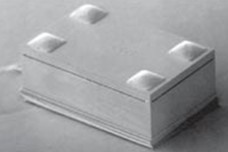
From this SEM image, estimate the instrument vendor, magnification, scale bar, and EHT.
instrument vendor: JEOL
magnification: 0.037 K X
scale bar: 1000000 nm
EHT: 10 kV